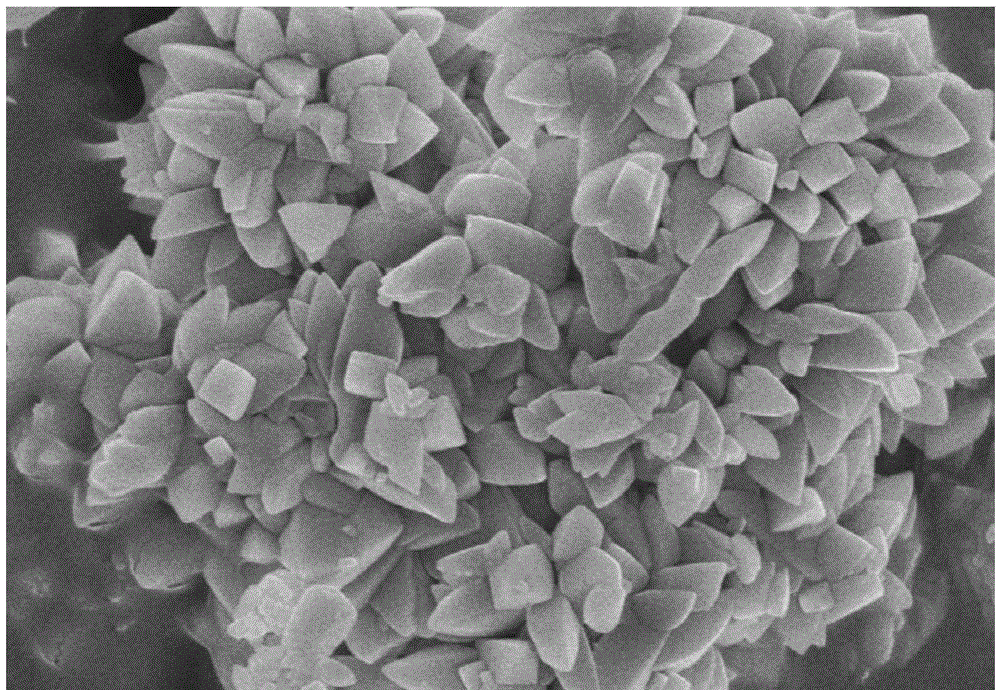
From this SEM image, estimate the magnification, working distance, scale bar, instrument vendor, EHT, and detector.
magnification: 30 K X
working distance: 7.6 mm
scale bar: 1000 nm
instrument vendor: Hitachi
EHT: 5 kV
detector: SE(U)